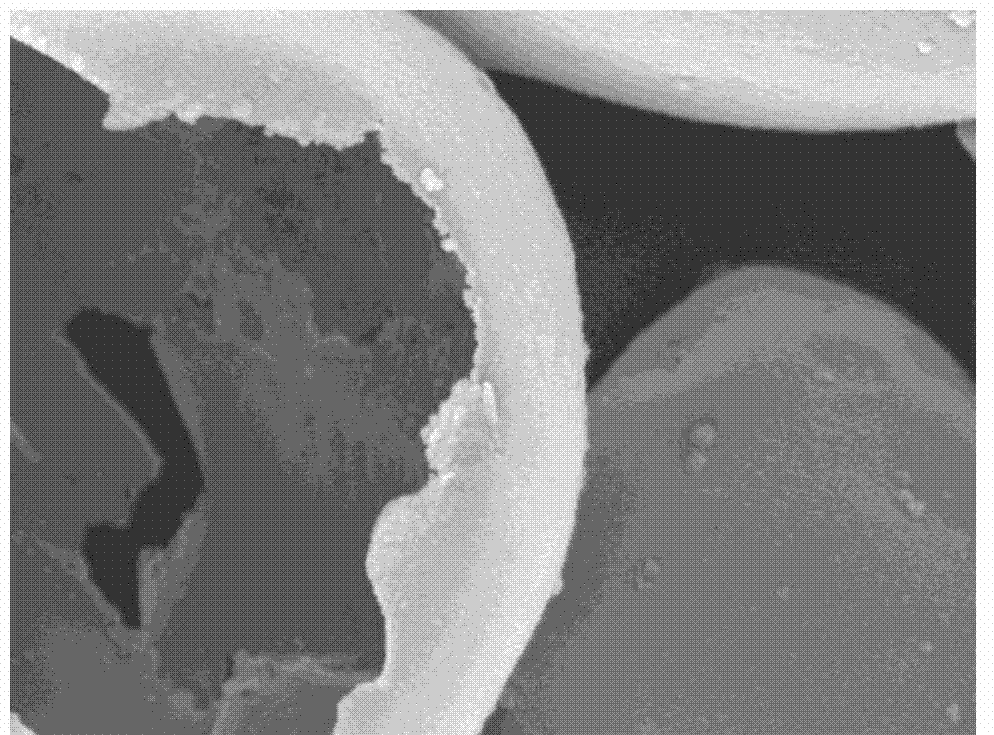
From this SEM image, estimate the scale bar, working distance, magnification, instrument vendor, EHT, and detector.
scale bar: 1000 nm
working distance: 15.1 mm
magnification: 10 K X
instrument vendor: JEOL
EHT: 20 kV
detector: LEI